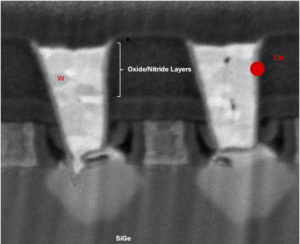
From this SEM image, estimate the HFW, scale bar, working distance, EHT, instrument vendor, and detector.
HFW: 0.32 µm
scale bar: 100 nm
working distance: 1.5 mm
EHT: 1 kV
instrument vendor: FEI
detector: BSE